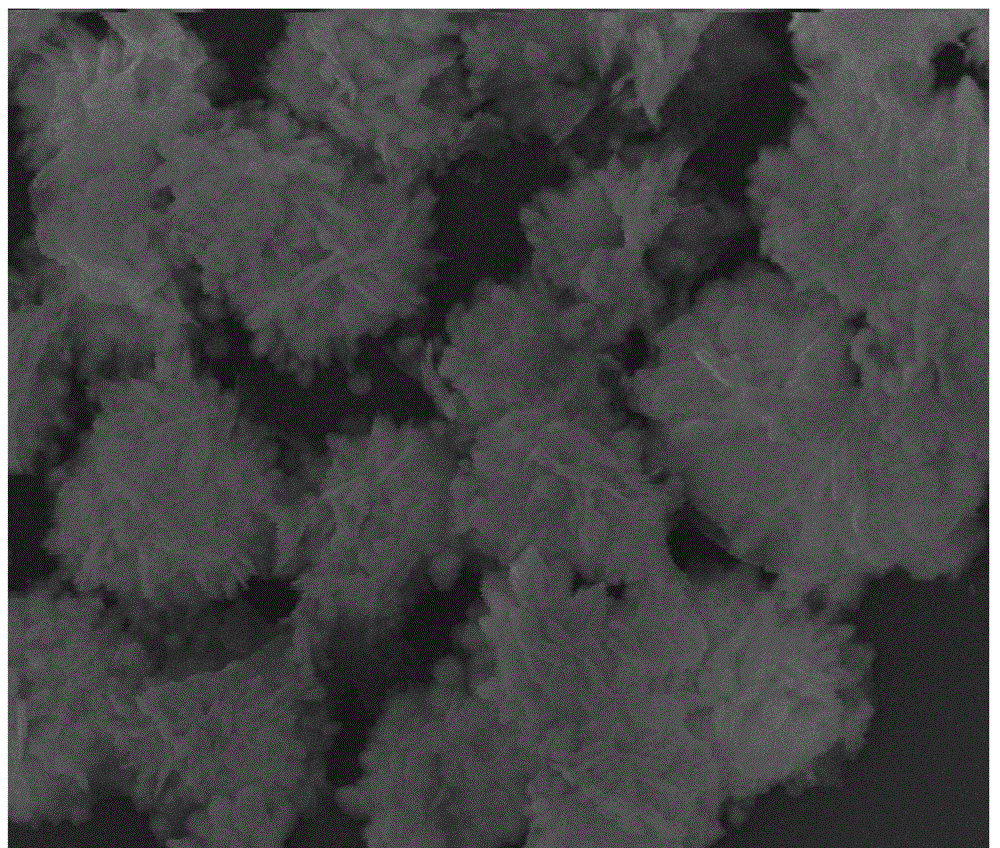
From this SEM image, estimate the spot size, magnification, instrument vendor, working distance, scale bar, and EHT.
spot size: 3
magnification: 50 K X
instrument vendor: FEI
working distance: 6.6 mm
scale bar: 2000 nm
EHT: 15 kV